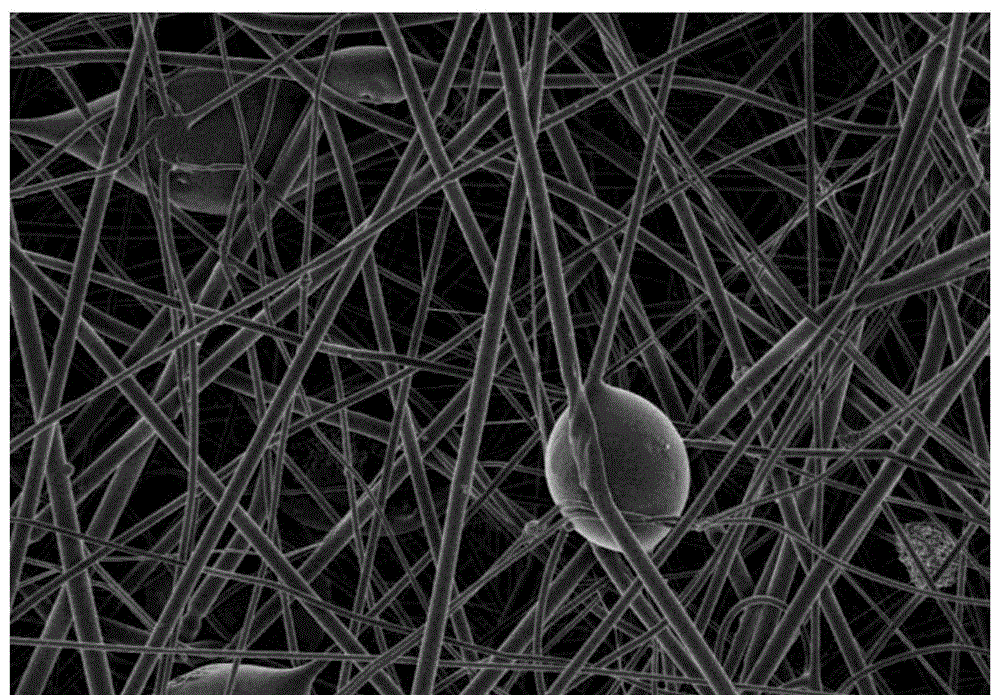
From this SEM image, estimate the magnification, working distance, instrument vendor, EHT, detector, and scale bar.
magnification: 5 K X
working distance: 3.4 mm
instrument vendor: Hitachi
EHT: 1.5 kV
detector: SE(U)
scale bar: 10000 nm